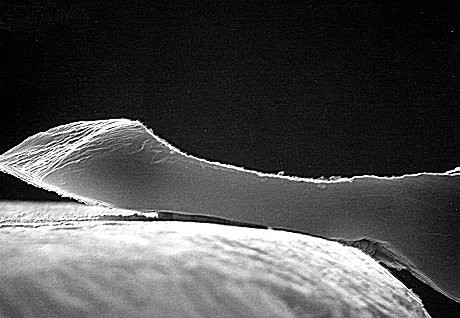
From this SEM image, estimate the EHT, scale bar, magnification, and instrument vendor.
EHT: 30 kV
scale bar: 10000 nm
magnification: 1.41 K X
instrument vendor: JEOL